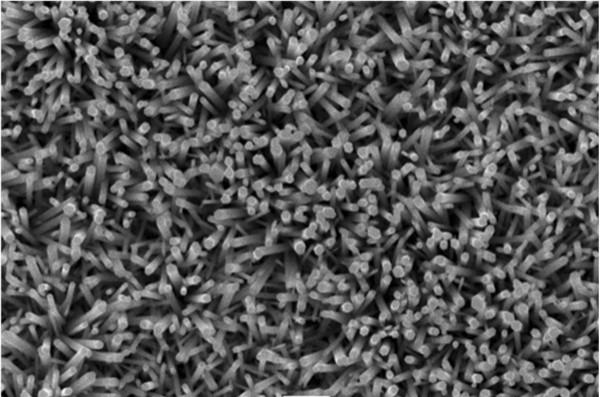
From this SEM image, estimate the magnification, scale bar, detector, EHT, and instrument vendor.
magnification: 10 K X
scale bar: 1000 nm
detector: SEI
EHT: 10 kV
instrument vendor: JEOL